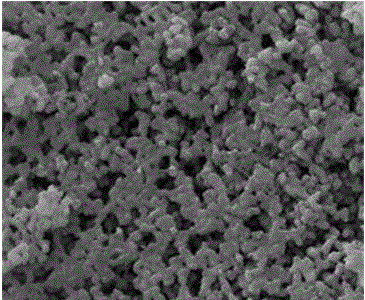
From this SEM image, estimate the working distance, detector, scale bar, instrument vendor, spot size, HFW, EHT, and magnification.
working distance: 8.6 mm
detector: ETD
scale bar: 500 nm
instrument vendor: FEI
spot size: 2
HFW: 2.98 µm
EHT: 10 kV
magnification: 50 K X